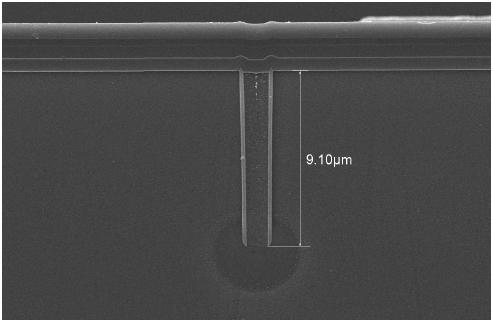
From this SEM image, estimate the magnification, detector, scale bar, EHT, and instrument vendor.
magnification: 5 K X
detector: SEI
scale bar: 5000 nm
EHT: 10 kV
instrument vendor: JEOL